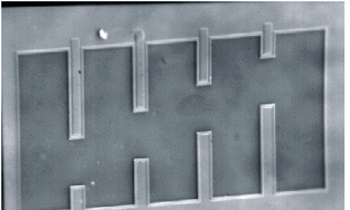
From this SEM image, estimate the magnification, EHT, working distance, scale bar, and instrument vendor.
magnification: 0.16 K X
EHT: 10 kV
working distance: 23 mm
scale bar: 100000 nm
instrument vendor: JEOL